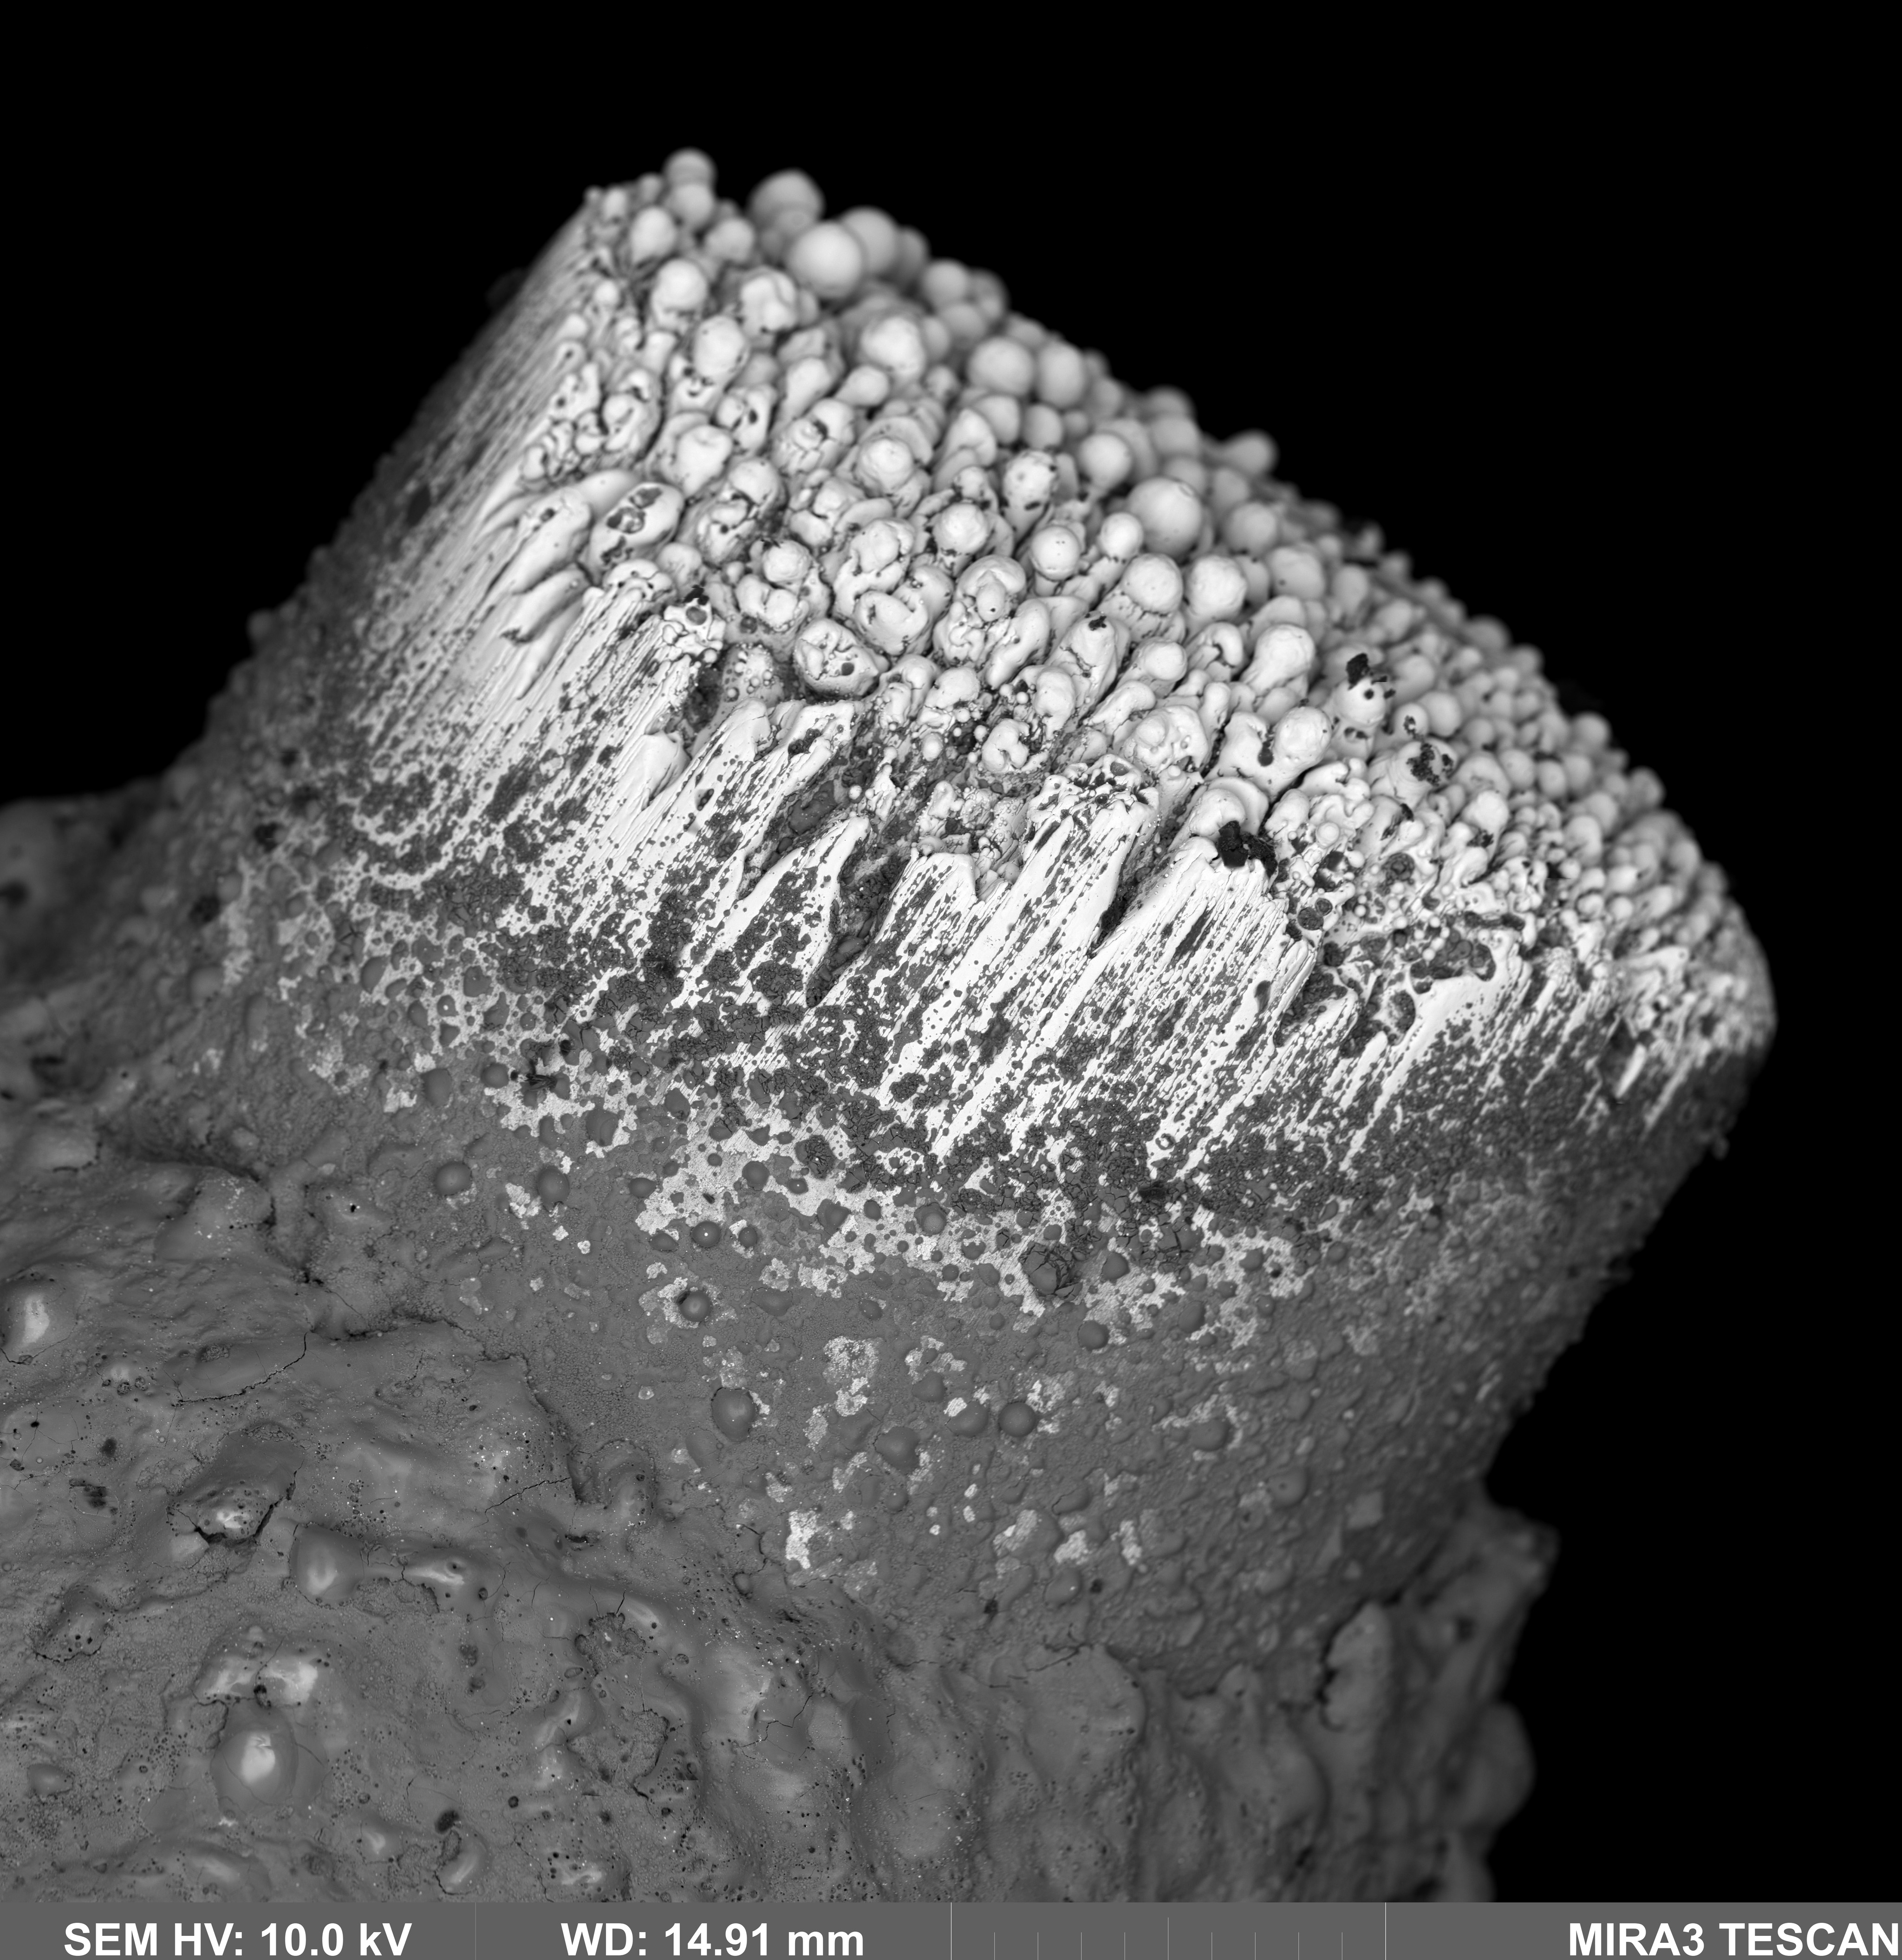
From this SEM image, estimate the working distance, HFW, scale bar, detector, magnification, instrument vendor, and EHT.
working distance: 14.91 mm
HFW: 875 µm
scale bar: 200000 nm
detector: LE-BSE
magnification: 2.56 K X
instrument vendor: Tescan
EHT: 10 kV